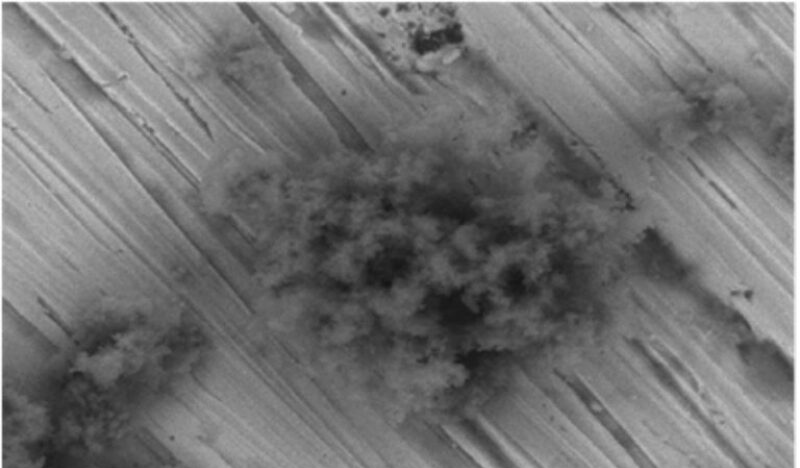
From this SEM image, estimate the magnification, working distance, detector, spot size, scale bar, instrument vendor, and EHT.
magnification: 2 K X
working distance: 10 mm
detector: BSE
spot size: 5.2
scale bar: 10000 nm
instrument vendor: FEI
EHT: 20 kV